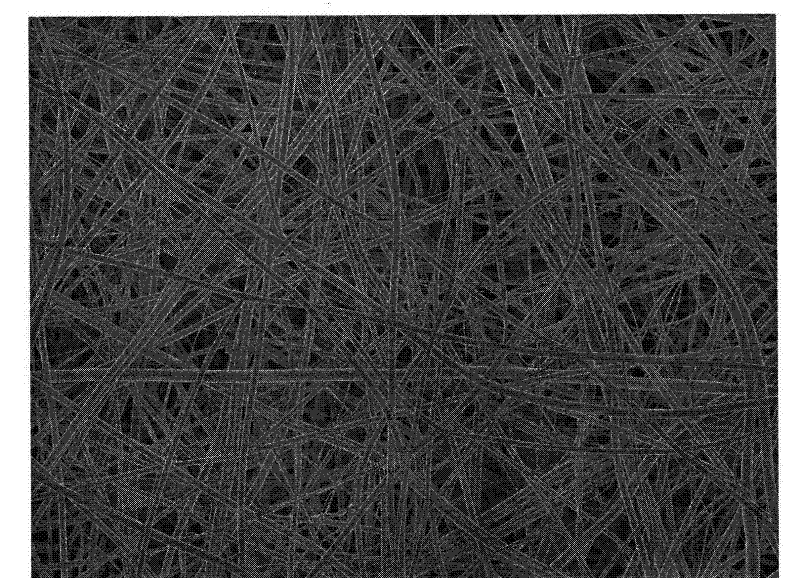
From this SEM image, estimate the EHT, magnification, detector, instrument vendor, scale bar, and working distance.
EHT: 3 kV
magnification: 1 K X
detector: SEI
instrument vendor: JEOL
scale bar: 10000 nm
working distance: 7 mm